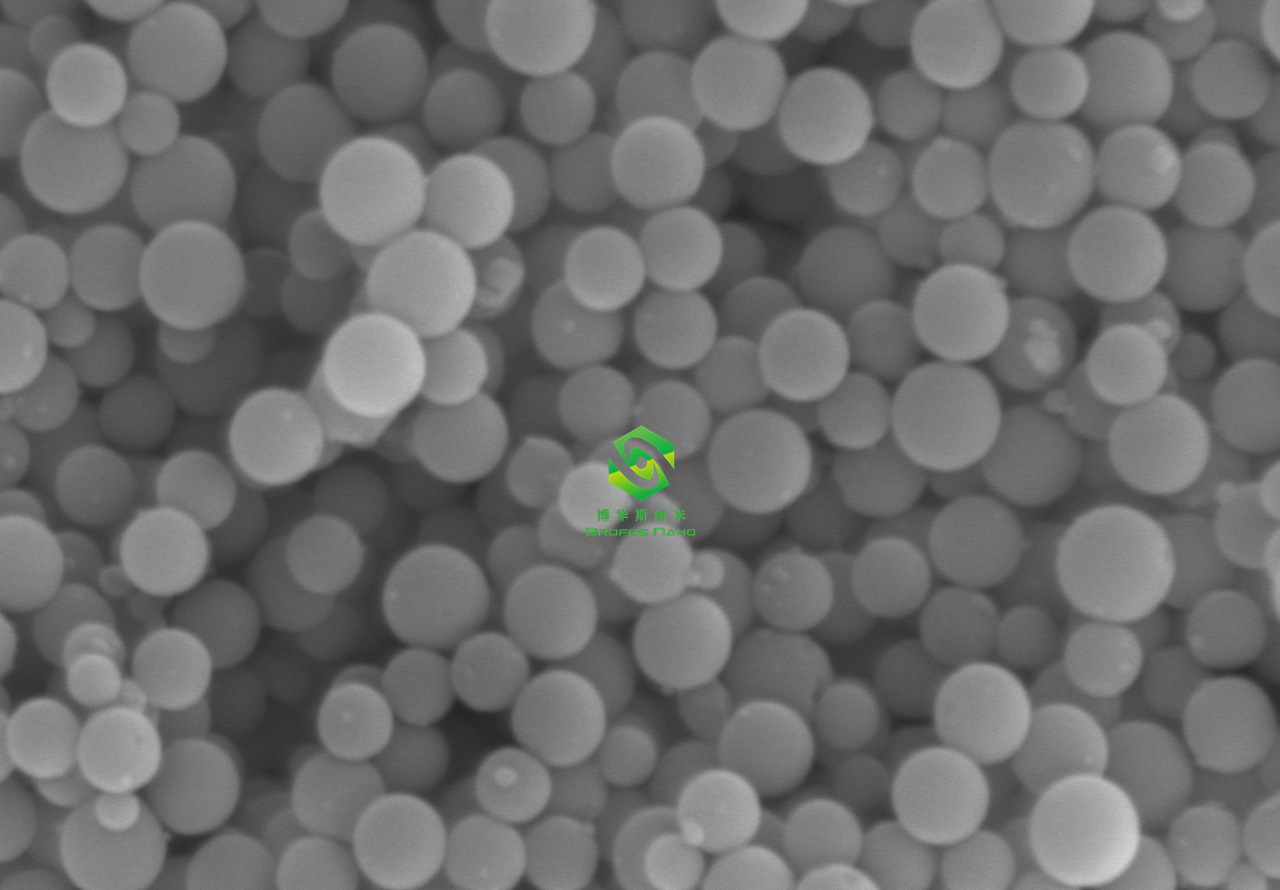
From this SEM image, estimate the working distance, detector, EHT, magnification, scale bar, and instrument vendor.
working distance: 8.5 mm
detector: SE(L)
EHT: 20 kV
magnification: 20 K X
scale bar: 2000 nm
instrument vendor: Hitachi